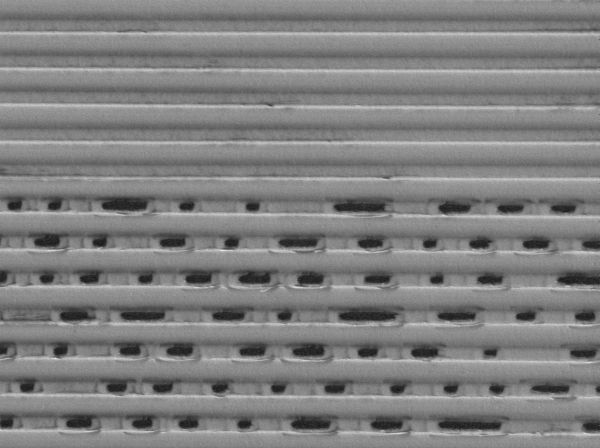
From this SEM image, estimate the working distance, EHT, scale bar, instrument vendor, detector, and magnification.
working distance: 7.9 mm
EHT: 2 kV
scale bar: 1000 nm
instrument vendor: JEOL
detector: LEI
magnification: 5 K X